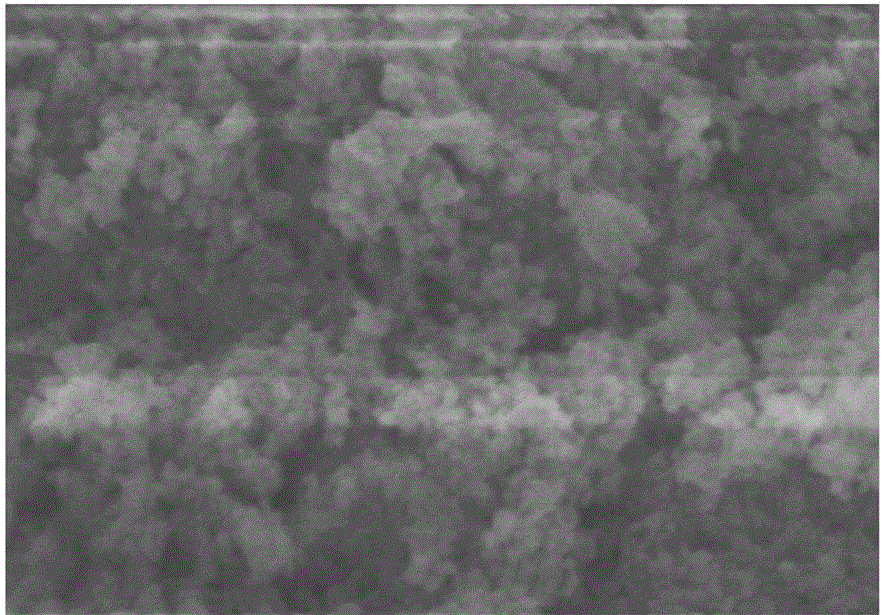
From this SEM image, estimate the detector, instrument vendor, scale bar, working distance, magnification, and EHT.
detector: SE(M)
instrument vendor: Hitachi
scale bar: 500 nm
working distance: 8.3 mm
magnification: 100 K X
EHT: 5 kV